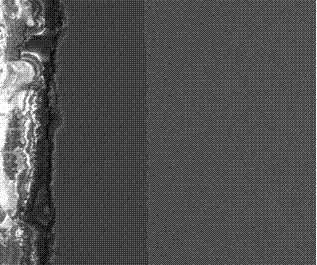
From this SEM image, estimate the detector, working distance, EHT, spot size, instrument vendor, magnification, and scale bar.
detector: ETD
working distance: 10.2 mm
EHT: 20 kV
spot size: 4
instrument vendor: FEI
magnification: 2 K X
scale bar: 50000 nm